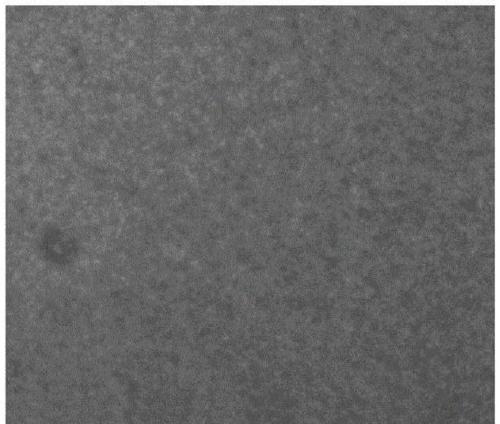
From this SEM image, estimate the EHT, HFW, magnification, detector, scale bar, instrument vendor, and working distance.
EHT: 10 kV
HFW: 138 µm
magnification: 1 K X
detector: SE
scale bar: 20000 nm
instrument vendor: Tescan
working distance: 8.21 mm